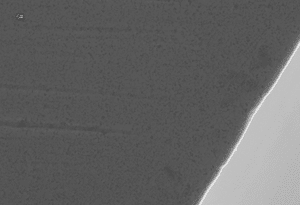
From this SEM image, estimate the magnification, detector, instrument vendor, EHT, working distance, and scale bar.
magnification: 3 K X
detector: SE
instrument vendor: Hitachi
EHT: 15 kV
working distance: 10.1 mm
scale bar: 10000 nm